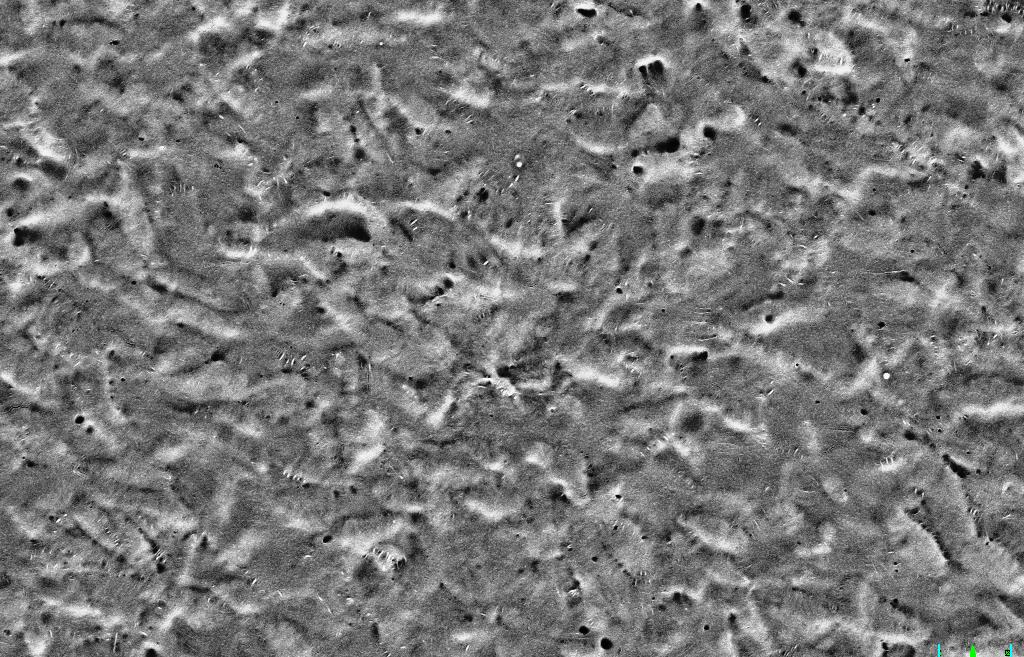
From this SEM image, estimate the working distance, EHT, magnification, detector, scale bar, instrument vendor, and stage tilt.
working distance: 3 mm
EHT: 5 kV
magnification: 20 K X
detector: SE2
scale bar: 1000 nm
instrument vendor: Zeiss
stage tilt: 54°